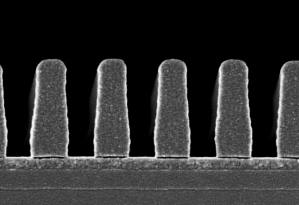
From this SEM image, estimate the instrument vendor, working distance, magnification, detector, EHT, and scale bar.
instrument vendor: Hitachi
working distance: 8.1 mm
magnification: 60 K X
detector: SE(U)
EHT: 1 kV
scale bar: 500 nm